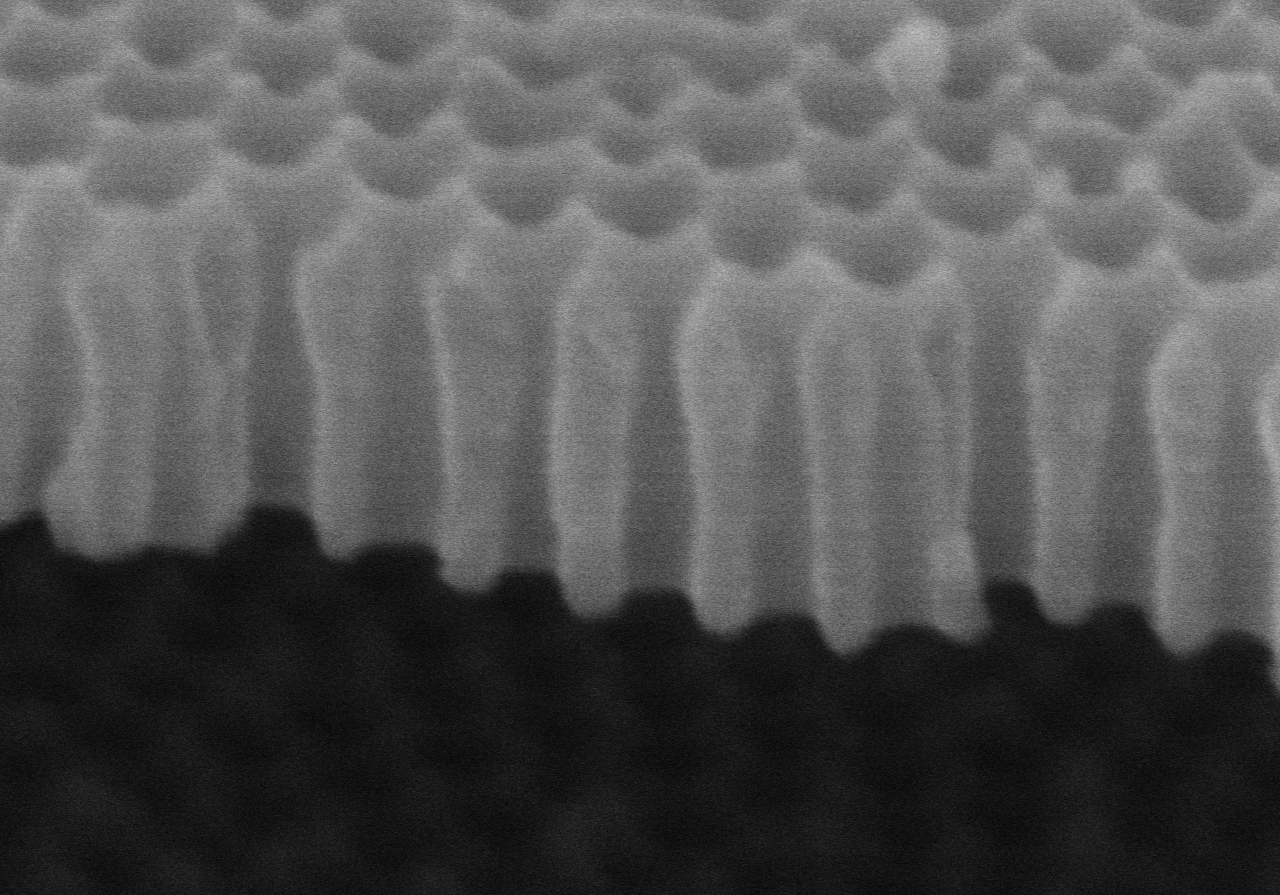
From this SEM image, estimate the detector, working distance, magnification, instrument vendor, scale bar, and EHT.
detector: SE(M,LA0)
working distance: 9.1 mm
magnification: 200 K X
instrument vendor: Hitachi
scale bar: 200 nm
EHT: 5 kV